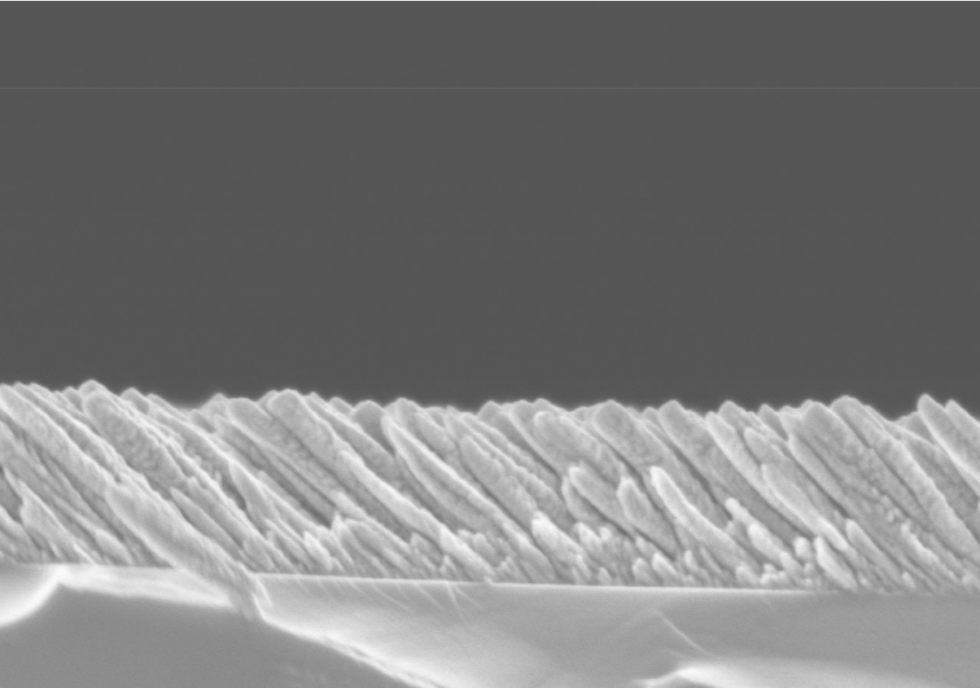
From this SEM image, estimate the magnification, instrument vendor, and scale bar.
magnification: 50 K X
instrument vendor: Hitachi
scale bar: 1000 nm